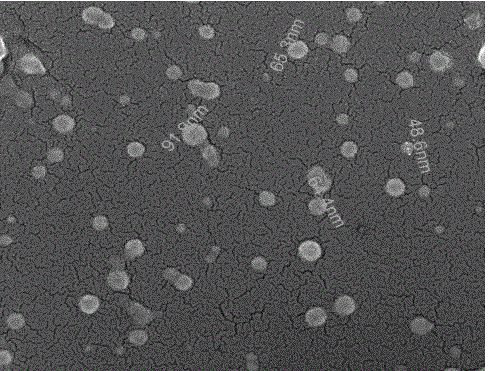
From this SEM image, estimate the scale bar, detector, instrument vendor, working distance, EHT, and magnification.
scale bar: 500 nm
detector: TLD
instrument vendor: FEI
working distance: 5.4 mm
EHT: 10 kV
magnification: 160 K X